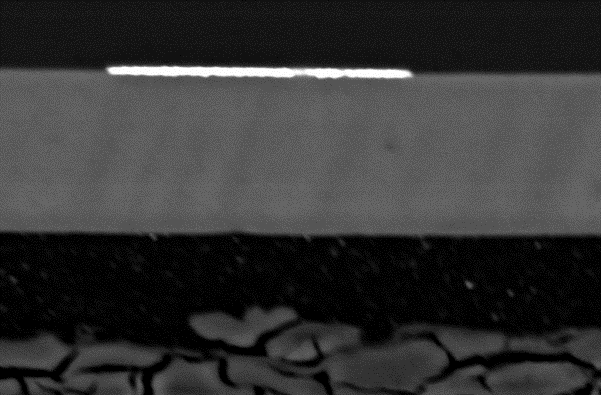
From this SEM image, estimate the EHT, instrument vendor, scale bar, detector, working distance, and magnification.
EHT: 20 kV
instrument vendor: Zeiss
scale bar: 20000 nm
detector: QBSD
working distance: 11 mm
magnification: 0.9 K X